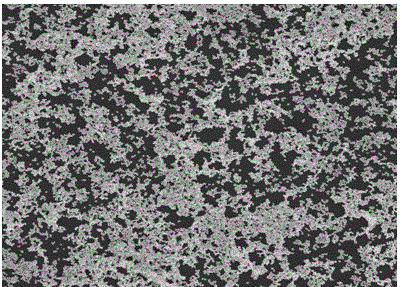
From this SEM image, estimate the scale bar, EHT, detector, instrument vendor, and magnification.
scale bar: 5000 nm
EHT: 5 kV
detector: TLD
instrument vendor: FEI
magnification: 30 K X